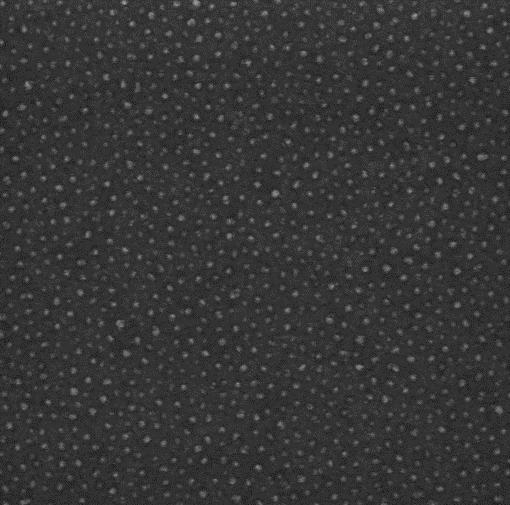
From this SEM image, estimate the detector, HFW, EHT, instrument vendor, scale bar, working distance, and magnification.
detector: InBeam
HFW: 2.889 µm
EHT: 15 kV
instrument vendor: Tescan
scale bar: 500 nm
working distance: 3.002 mm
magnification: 100 K X